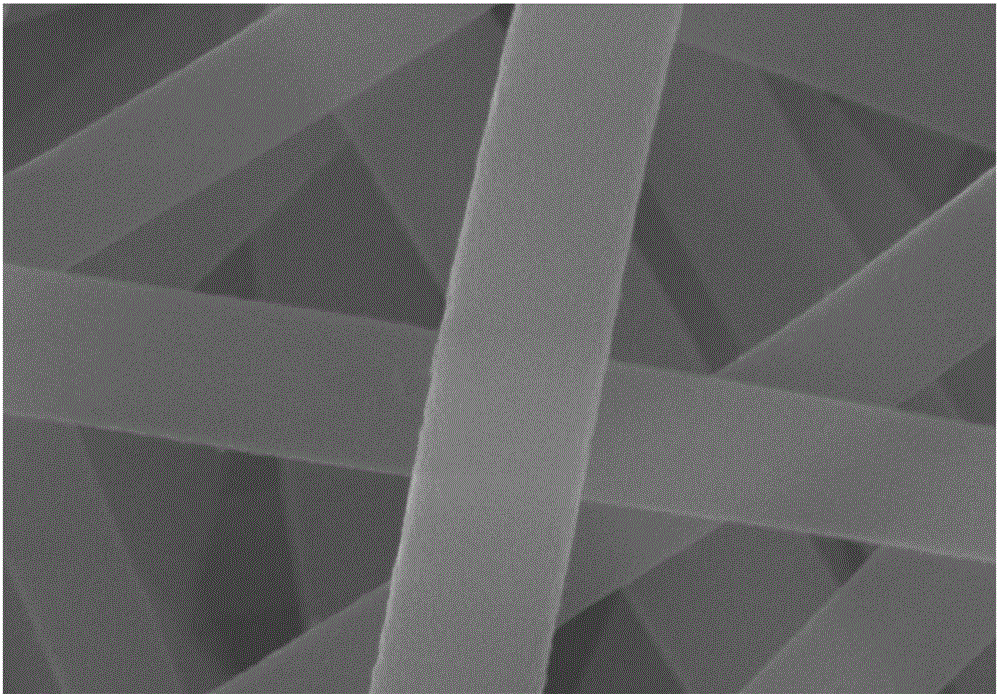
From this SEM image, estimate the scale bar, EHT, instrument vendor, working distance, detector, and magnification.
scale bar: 200 nm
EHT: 20 kV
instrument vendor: Zeiss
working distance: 5.8 mm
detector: InLens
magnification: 200 K X